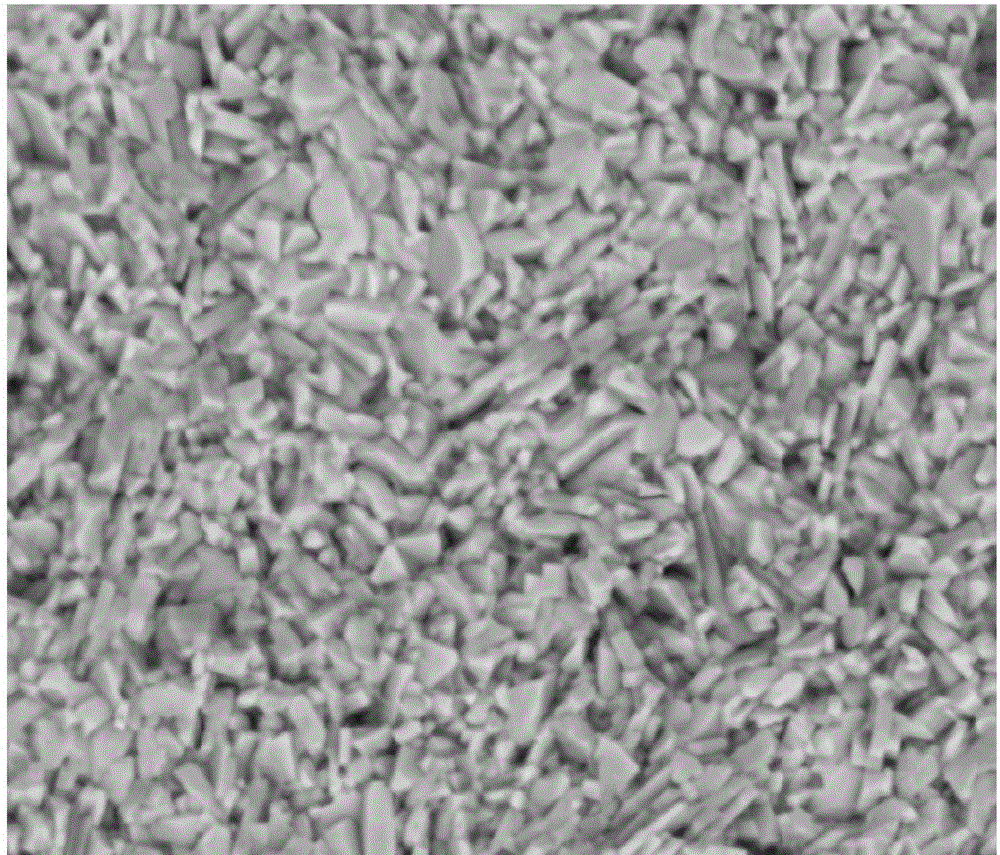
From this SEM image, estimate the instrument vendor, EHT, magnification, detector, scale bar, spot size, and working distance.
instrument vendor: FEI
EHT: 20 kV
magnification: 10 K X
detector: SSD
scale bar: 5000 nm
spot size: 4.5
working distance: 10 mm